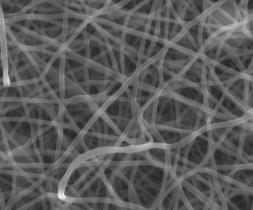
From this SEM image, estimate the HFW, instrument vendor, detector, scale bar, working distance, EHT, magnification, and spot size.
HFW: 9.95 µm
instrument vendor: FEI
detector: LVD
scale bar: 3000 nm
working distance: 5.9 mm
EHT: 18 kV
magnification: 20 K X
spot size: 4